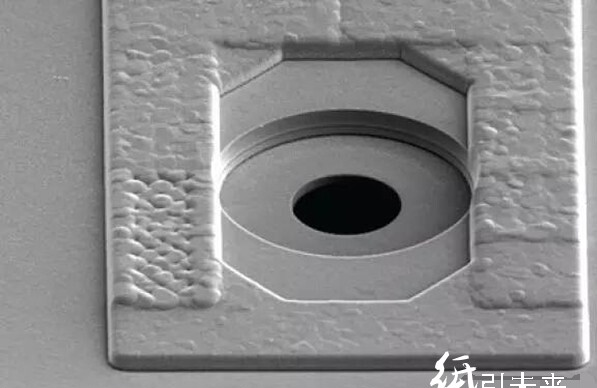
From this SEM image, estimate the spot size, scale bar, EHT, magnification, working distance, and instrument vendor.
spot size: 3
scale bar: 10000 nm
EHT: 5 kV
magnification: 2 K X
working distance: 17.1 mm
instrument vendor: FEI